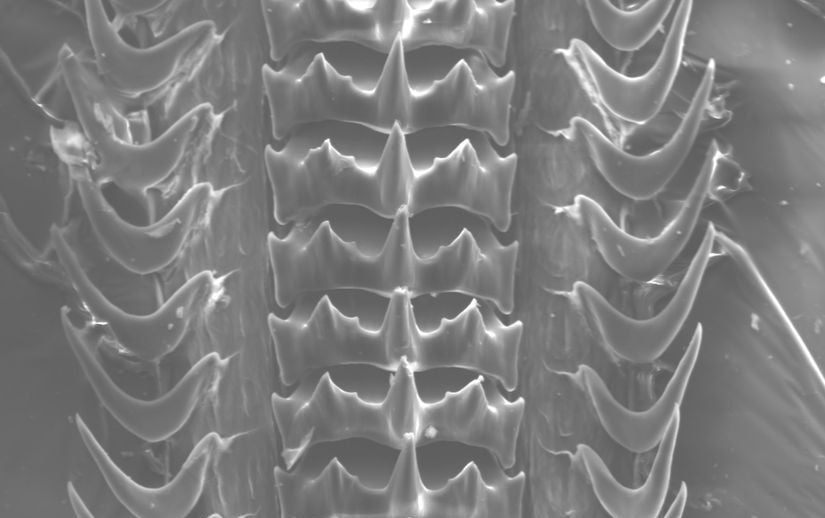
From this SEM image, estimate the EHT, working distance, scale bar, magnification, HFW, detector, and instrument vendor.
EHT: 20 kV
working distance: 17 mm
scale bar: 100000 nm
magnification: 0.437 K X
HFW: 631.6 µm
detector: SE1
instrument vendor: Zeiss